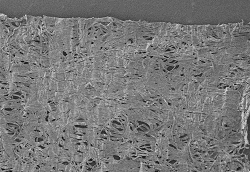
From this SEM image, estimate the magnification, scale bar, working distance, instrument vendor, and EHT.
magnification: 0.03 K X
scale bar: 1000000 nm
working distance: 9.2 mm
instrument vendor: Hitachi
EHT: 3 kV